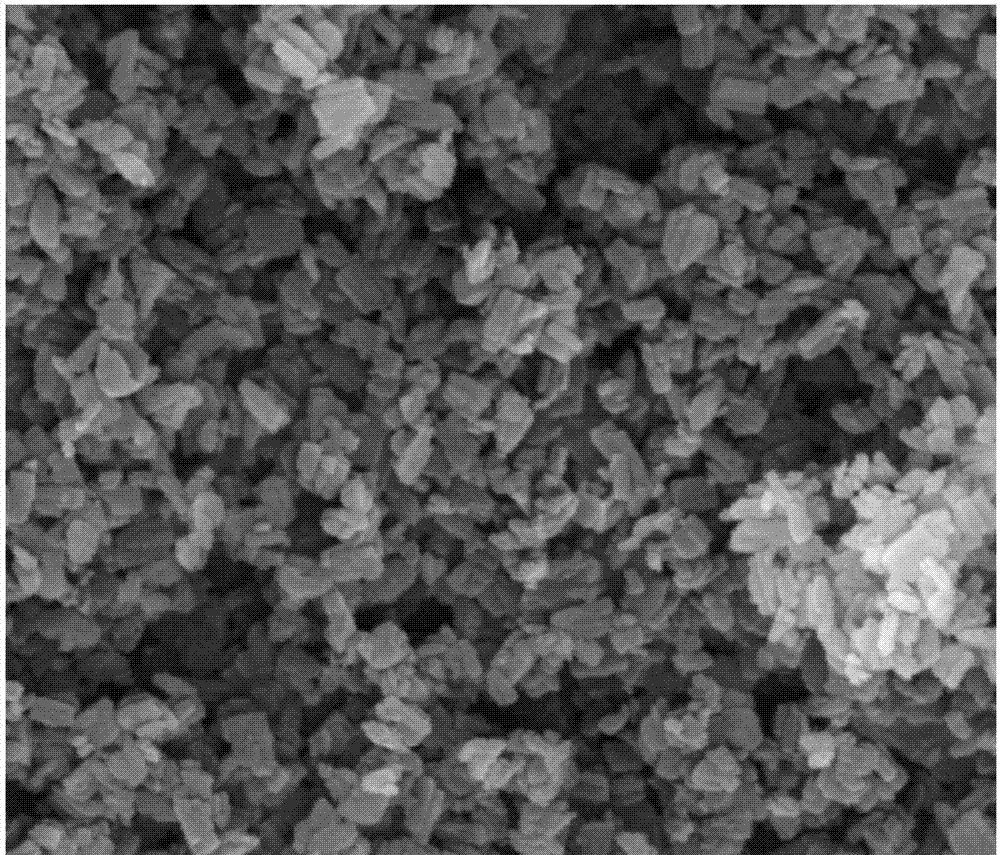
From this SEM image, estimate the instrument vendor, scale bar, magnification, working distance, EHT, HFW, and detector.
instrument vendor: FEI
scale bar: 1000 nm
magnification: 60 K X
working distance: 9.9 mm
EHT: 20 kV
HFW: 4.97 µm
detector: ETD